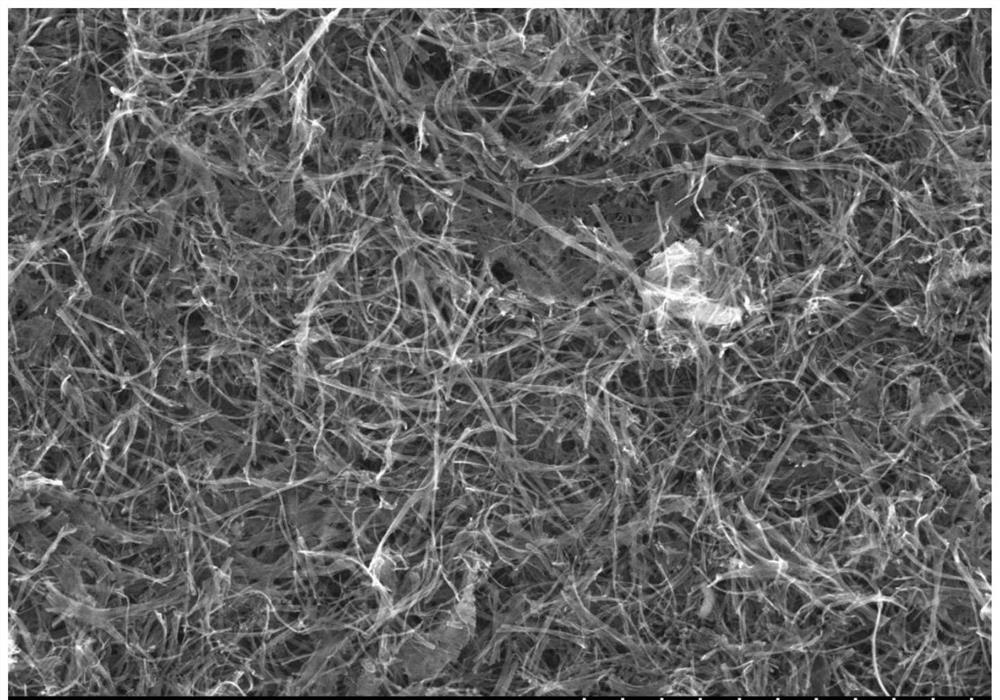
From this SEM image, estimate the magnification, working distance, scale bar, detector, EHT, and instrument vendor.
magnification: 10 K X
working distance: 7.3 mm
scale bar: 5000 nm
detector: SE(M)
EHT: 10 kV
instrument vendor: Hitachi